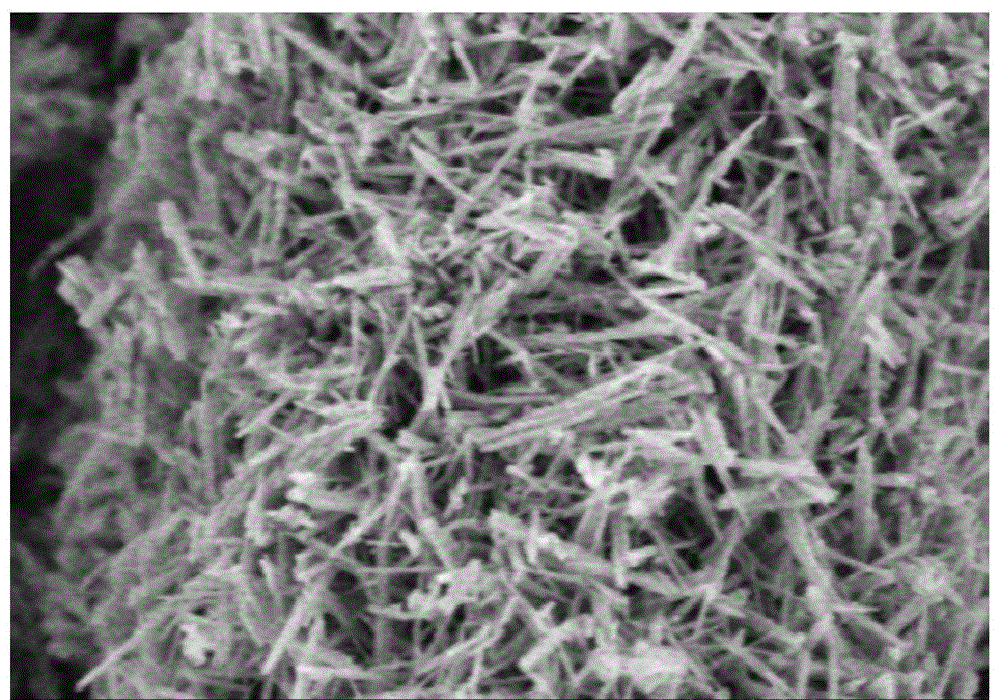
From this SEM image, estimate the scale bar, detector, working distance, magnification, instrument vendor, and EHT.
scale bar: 500 nm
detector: SE(M)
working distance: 7.4 mm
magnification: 80 K X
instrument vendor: Hitachi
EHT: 3 kV